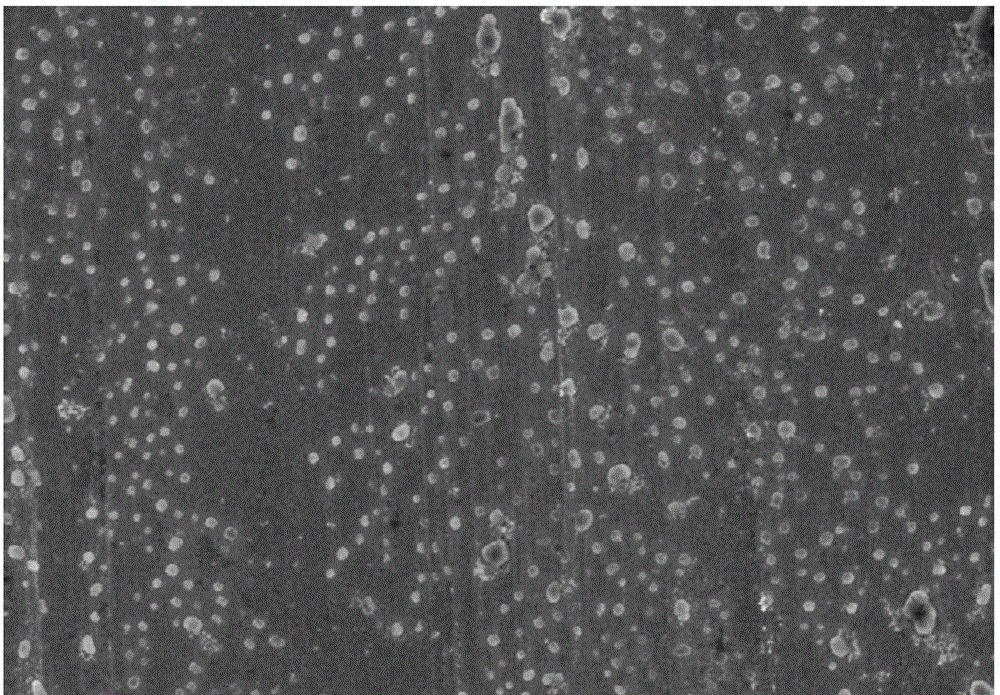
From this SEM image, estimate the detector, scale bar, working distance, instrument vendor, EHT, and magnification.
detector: SE(M)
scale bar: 5000 nm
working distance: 9.4 mm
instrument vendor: Hitachi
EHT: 5 kV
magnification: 6 K X